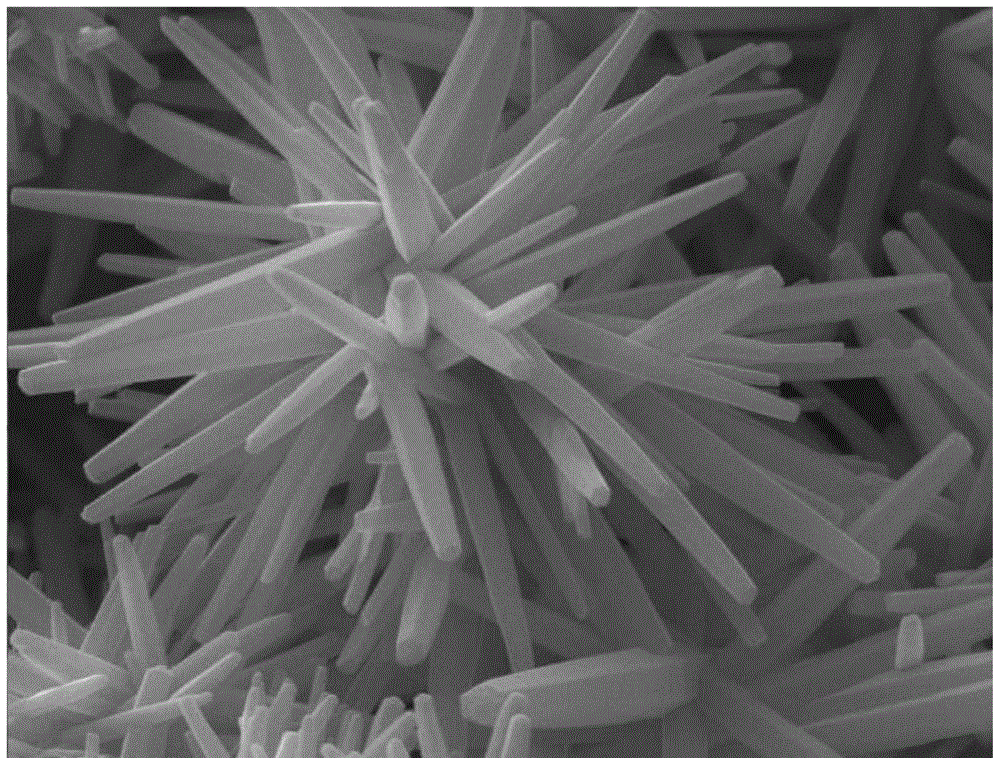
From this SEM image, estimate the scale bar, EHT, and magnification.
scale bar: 1000 nm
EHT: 15 kV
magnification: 20 K X